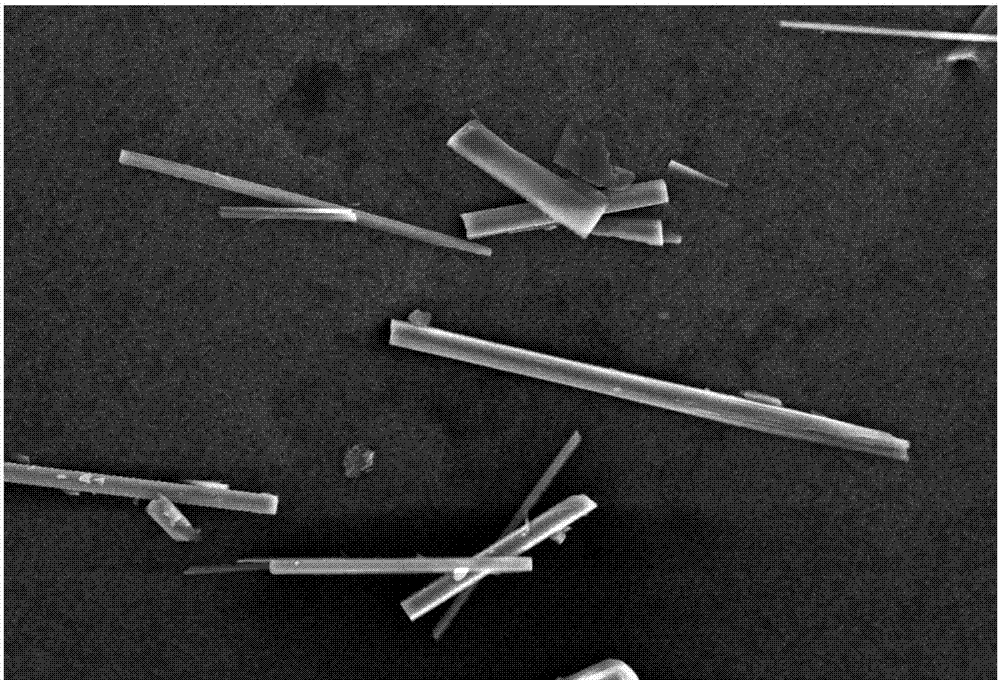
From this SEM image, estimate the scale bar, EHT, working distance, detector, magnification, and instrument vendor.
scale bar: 50000 nm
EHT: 20 kV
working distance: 16 mm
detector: SEI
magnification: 0.4 K X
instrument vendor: JEOL